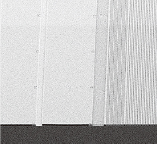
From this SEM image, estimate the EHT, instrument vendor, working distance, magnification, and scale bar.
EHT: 5 kV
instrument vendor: Hitachi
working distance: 5.8 mm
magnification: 0.13 K X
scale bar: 400000 nm